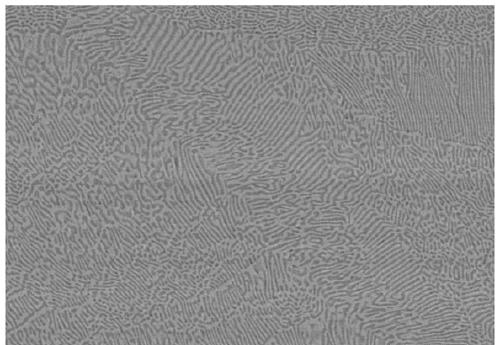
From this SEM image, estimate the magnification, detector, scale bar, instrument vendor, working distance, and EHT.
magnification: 1 K X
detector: SE(M,LA50)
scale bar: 50000 nm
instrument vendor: Hitachi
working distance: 8.5 mm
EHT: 25 kV